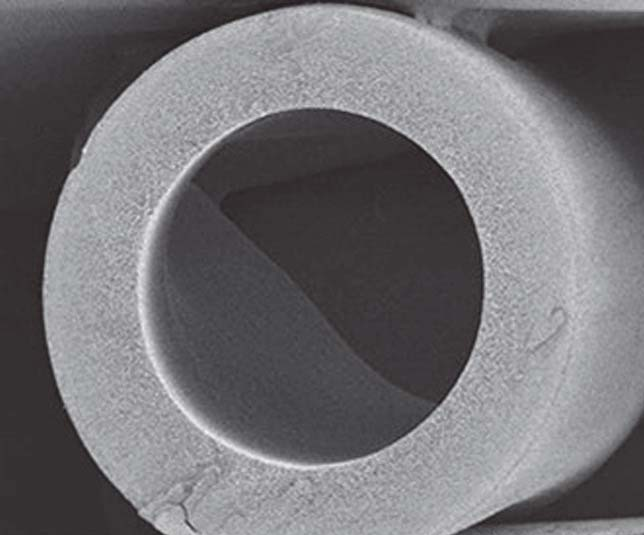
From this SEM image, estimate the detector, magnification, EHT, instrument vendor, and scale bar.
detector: SE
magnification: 0.2 K X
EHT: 1 kV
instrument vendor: Hitachi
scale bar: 200000 nm